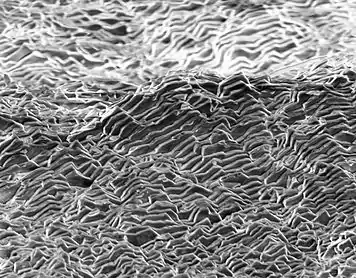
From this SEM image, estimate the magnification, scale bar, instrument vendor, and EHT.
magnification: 1.3 K X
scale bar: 20000 nm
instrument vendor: other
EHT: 10 kV